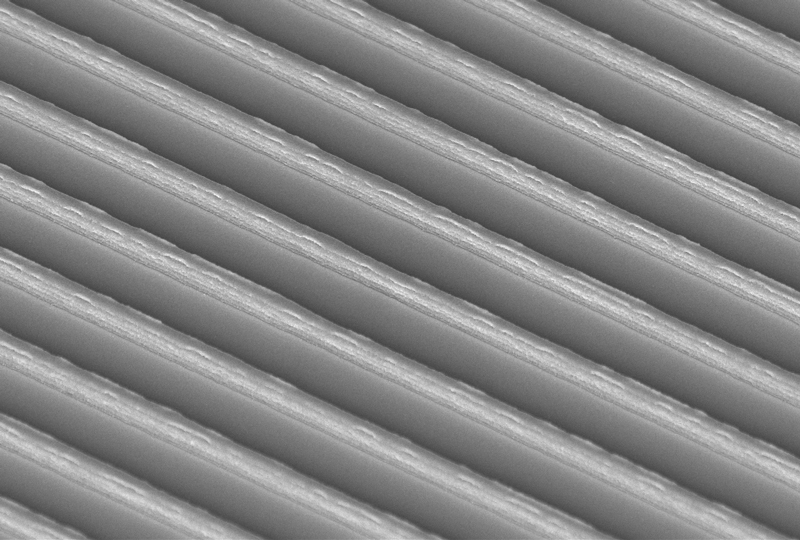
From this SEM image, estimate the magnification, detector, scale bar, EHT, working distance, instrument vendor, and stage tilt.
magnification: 7 K X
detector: InLens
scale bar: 1000 nm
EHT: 3 kV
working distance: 7 mm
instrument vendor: Zeiss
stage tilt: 45°